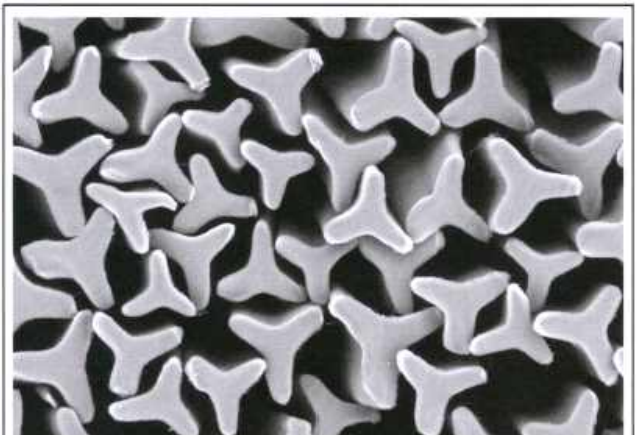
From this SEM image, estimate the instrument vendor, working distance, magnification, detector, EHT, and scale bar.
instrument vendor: Hitachi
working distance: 6.4 mm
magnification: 0.3 K X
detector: BSE-COMP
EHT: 20 kV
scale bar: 100000 nm